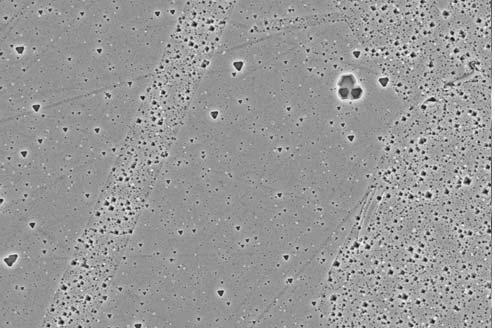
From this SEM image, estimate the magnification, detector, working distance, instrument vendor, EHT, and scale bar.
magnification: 2.5 K X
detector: InLens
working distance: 3.5 mm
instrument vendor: Zeiss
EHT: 15 kV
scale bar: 2000 nm